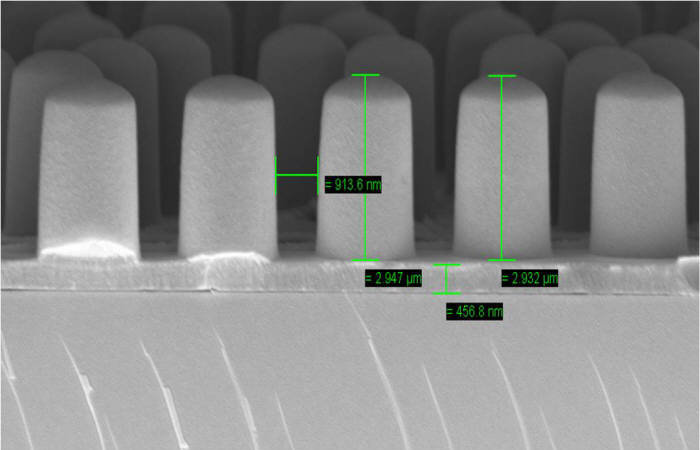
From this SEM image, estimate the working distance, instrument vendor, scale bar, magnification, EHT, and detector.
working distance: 8.5 mm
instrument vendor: Zeiss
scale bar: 1000 nm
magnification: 23.59 K X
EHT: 5 kV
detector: InLens